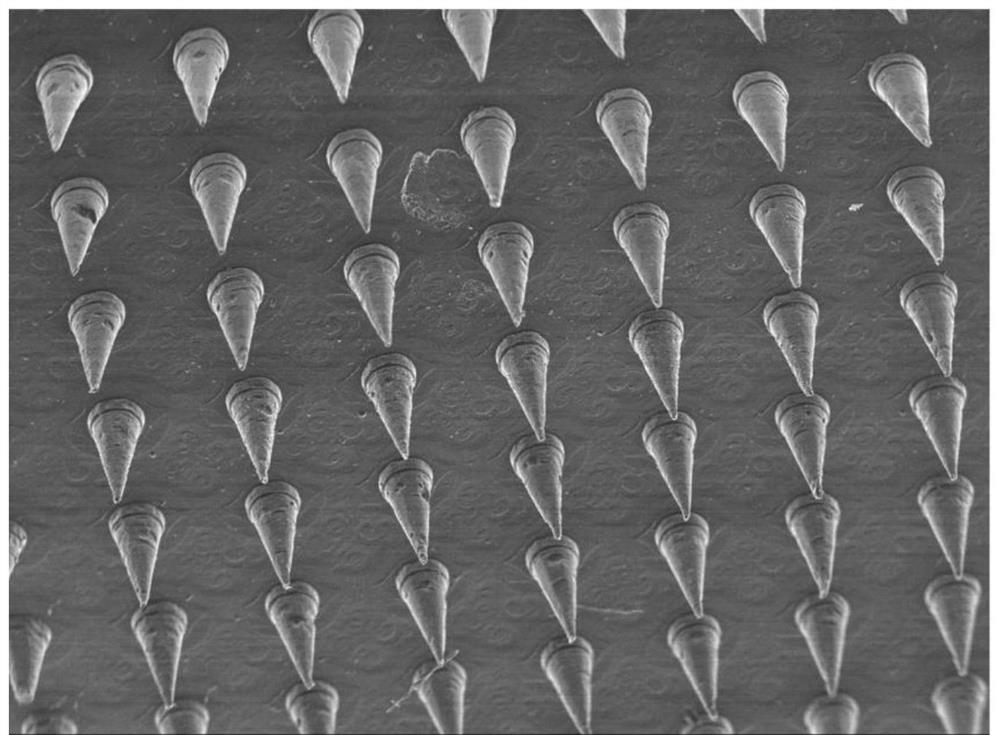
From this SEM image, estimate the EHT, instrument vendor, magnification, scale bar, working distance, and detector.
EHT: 15 kV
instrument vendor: JEOL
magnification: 0.02 K X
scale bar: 1000000 nm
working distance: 18 mm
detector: SEI